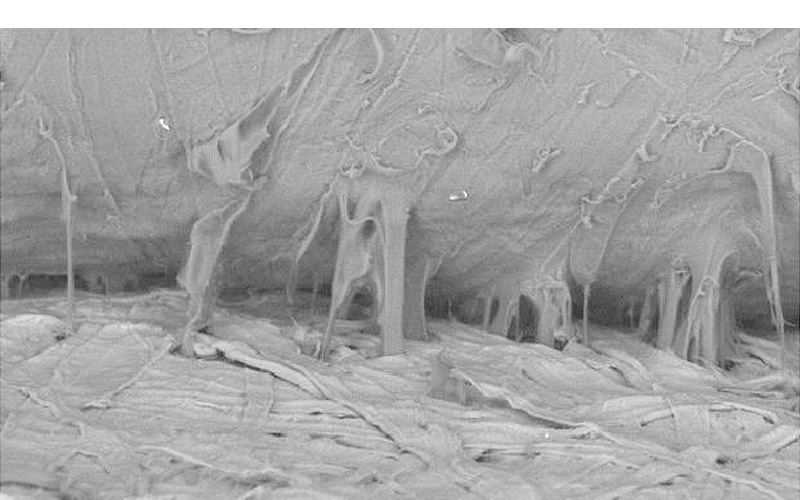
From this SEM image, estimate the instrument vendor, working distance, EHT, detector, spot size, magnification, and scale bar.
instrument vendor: FEI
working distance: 16.3 mm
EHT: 15 kV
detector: BSE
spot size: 6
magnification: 0.261 K X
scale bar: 100000 nm